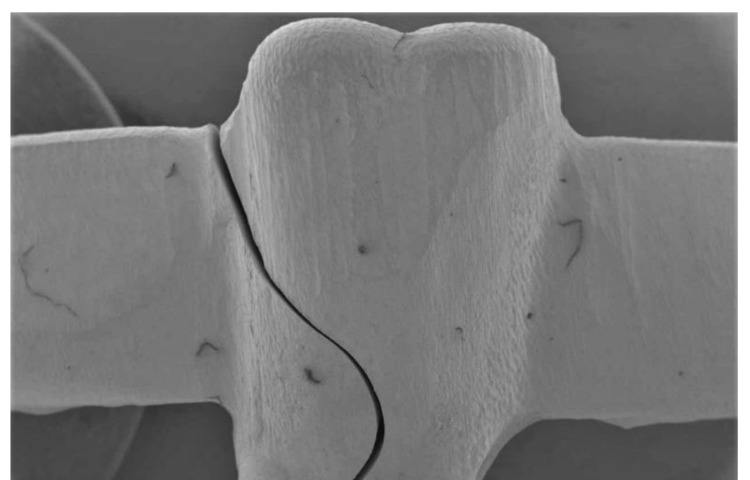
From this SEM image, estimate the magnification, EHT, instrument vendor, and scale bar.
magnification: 0.01 K X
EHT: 10 kV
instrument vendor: JEOL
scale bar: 2000000 nm